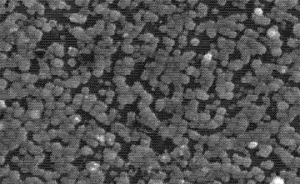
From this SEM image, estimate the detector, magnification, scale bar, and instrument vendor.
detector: SE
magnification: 15 K X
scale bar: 3000 nm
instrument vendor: Hitachi